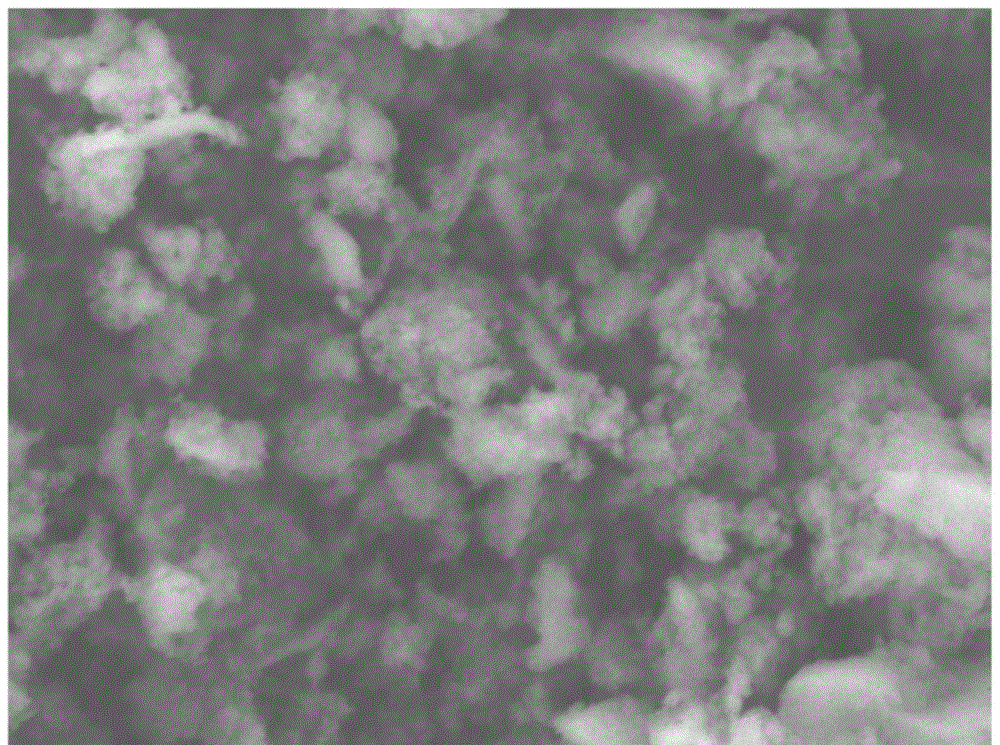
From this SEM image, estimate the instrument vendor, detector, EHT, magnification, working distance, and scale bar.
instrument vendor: JEOL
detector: LEI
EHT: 5 kV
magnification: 30 K X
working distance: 7.8 mm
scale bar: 100 nm